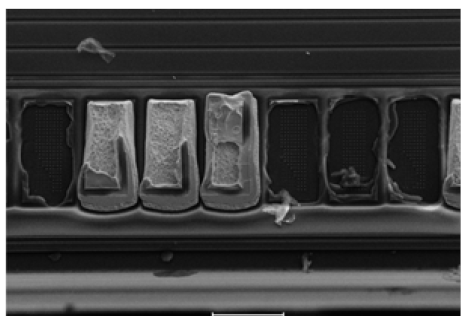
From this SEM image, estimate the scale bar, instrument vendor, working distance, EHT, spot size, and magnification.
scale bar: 60000 nm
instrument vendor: other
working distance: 6 mm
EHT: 20 kV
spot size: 10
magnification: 0.5 K X